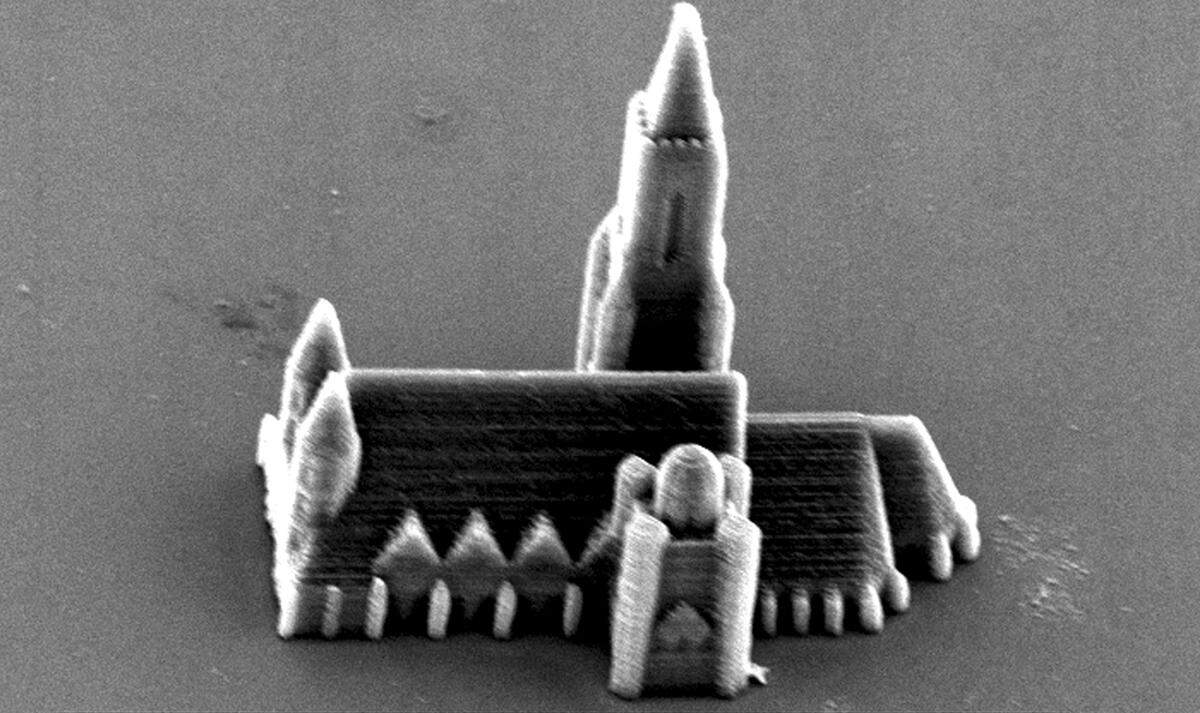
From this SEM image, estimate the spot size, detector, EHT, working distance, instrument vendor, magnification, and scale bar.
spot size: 4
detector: SE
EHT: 15 kV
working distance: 27.5 mm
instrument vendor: FEI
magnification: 0.859 K X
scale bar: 20000 nm